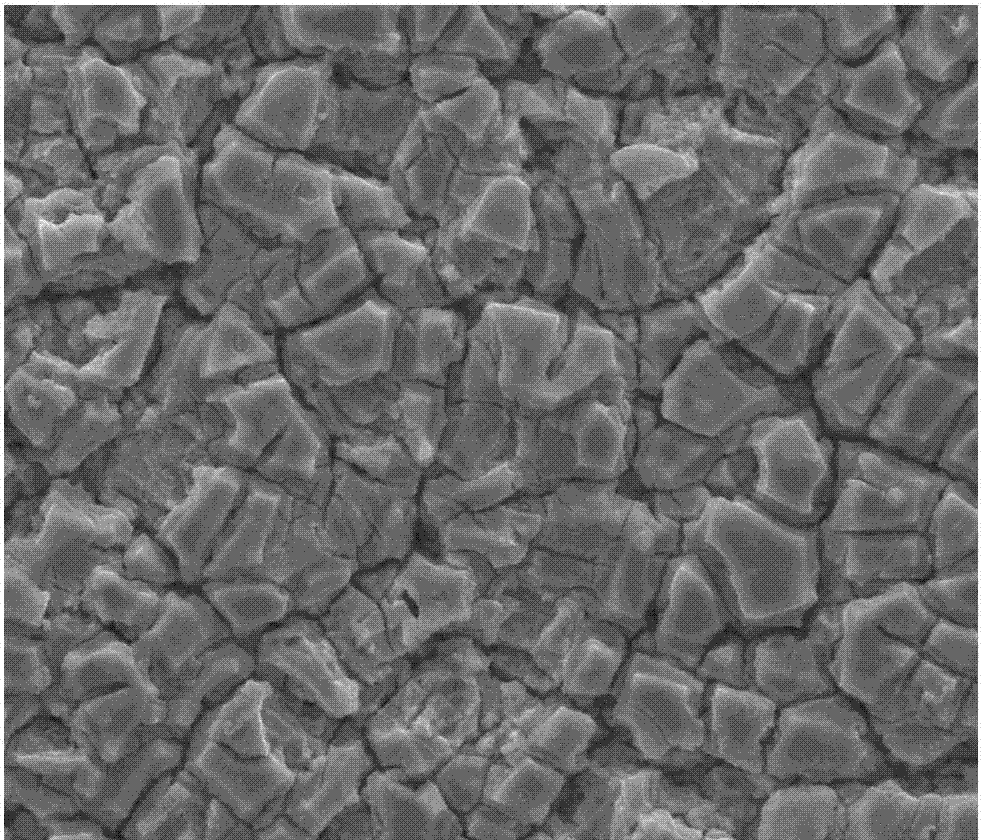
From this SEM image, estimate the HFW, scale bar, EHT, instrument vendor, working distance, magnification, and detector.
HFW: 67.6 µm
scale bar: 10000 nm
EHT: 20 kV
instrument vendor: FEI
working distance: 10.2 mm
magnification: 2 K X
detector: ETD